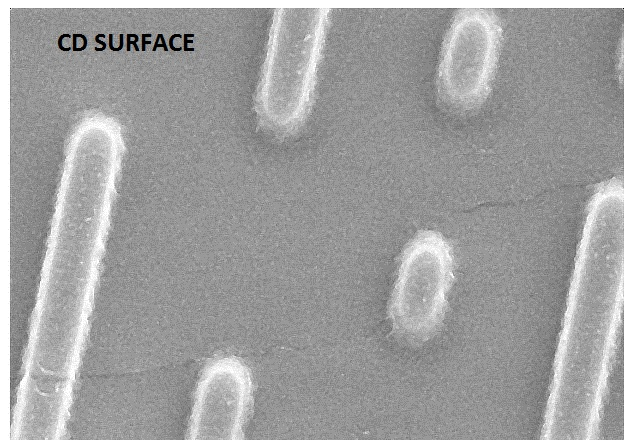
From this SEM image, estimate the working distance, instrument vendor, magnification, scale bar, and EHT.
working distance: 6 mm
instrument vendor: JEOL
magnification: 20 K X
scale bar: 2000 nm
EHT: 5 kV